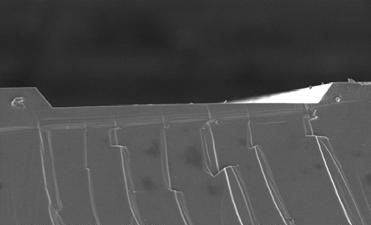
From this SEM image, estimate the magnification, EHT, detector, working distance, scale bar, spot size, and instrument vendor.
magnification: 0.25 K X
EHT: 15 kV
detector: SE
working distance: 10.4 mm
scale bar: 100000 nm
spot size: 3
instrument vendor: FEI